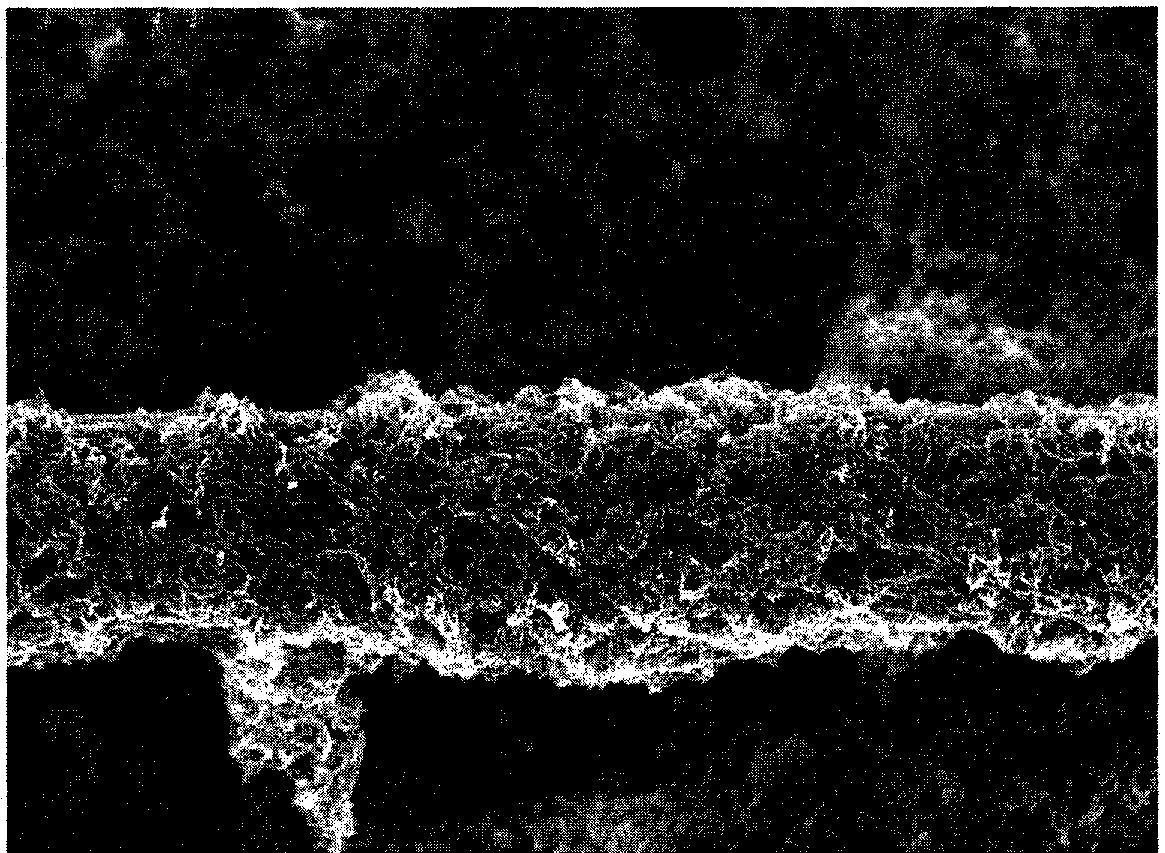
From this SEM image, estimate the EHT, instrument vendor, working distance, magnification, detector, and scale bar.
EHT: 10 kV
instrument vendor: Zeiss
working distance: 8.2 mm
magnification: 2.5 K X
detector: InLens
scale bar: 2000 nm